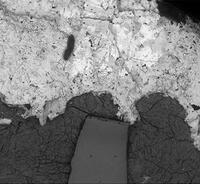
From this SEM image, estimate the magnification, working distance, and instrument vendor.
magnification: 0.06 K X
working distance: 9.6 mm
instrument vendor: Hitachi